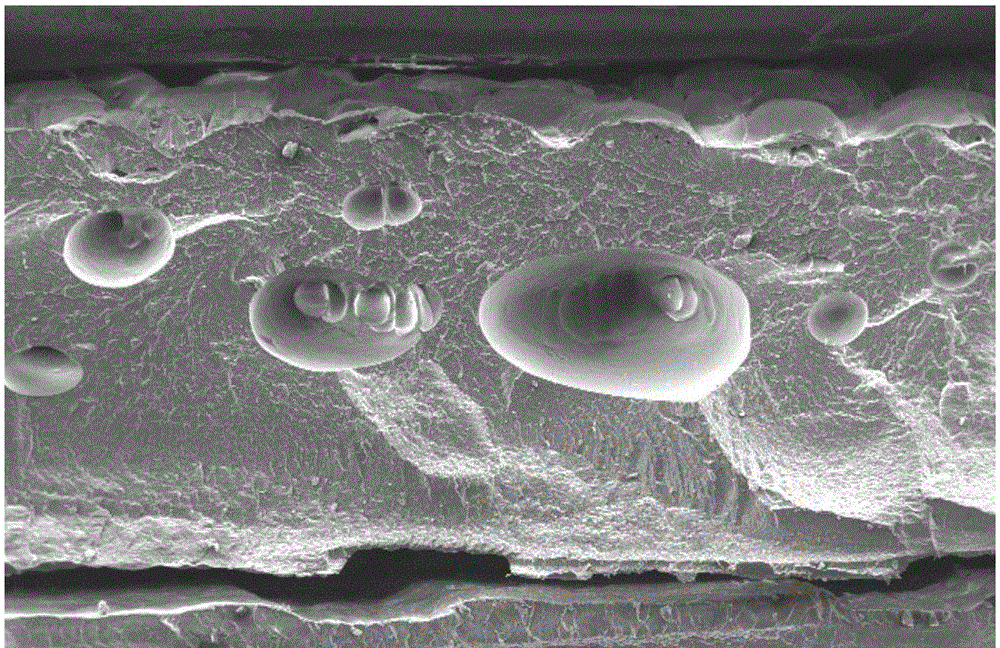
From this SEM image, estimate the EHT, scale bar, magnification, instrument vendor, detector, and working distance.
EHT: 15 kV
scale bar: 1000000 nm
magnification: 0.03 K X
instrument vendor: FEI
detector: SE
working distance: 29.9 mm